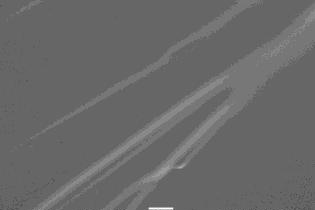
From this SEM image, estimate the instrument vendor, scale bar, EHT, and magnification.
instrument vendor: JEOL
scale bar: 10000 nm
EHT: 15 kV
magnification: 1 K X